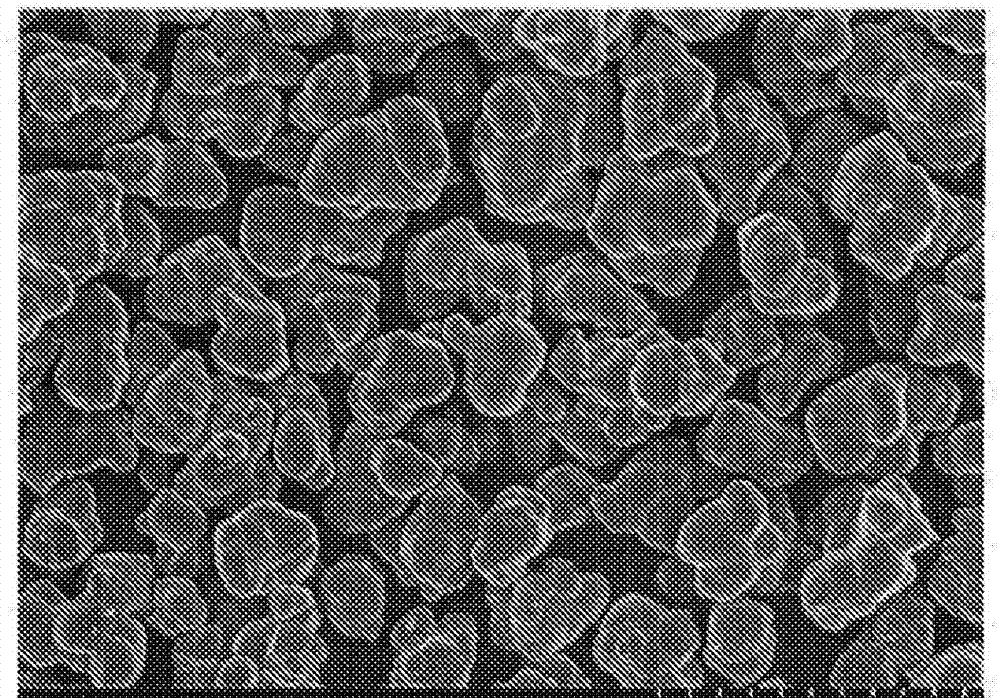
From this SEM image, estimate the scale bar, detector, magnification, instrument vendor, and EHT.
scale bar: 20000 nm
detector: SE(U)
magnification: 2 K X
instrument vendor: Hitachi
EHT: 10 kV